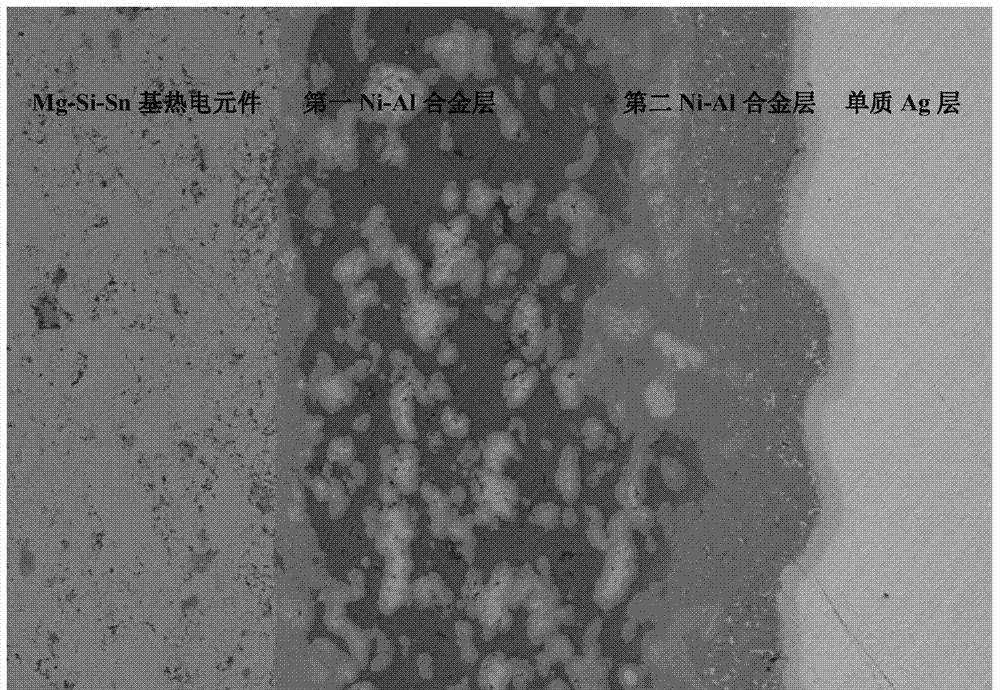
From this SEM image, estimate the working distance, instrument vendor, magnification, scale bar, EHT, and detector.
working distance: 15.3 mm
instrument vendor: Hitachi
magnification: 0.12 K X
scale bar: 400000 nm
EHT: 10 kV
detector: LM(UL)